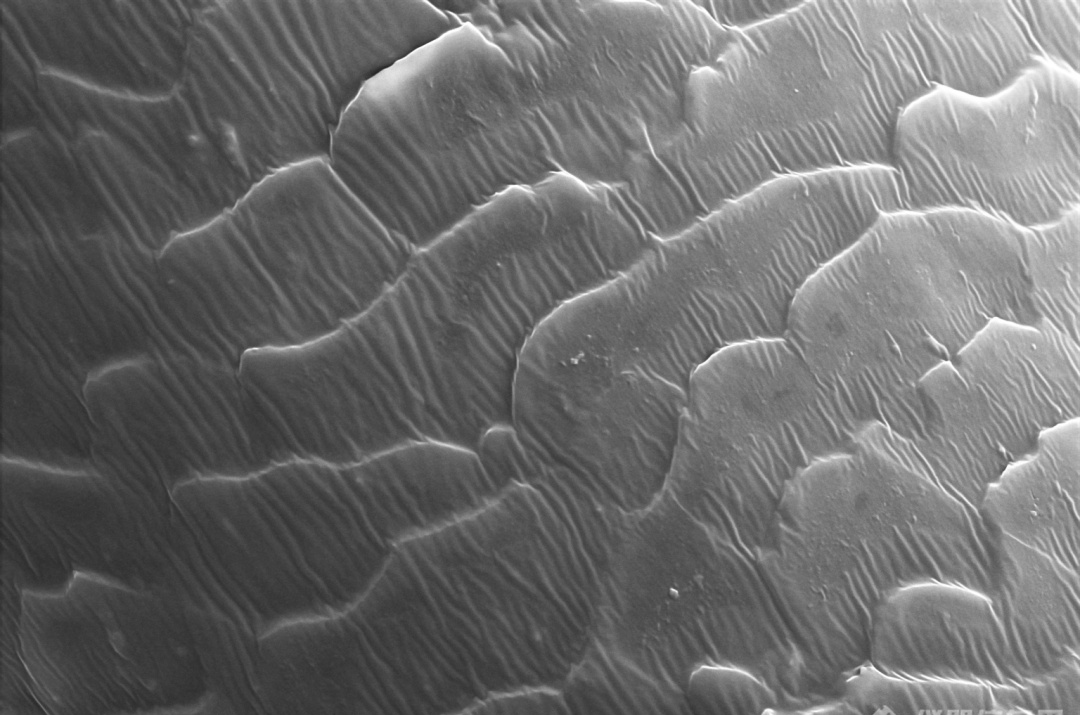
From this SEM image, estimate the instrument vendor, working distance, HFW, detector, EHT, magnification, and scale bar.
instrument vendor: Thermo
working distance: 8.1 mm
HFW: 63.5 µm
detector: ETD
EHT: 15 kV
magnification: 2 K X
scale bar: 10000 nm